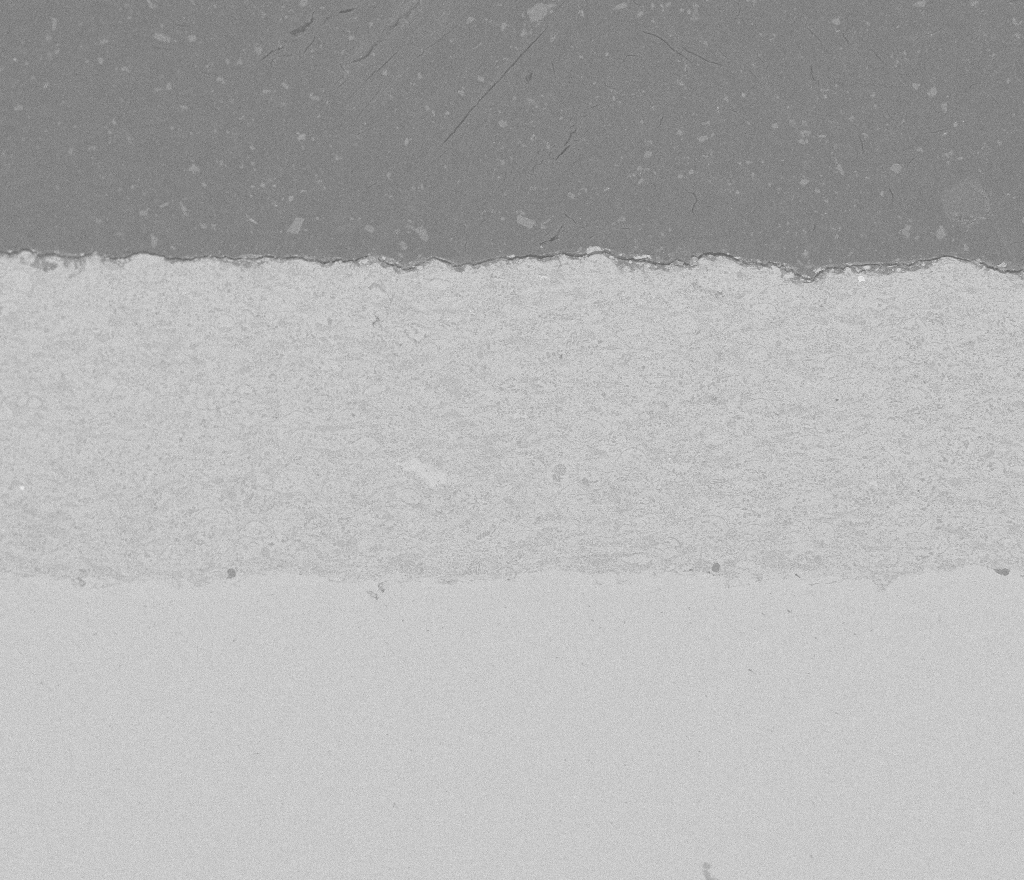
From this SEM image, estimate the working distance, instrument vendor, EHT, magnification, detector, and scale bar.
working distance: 16.3 mm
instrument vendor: FEI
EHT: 15 kV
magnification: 0.25 K X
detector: vCD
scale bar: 500000 nm